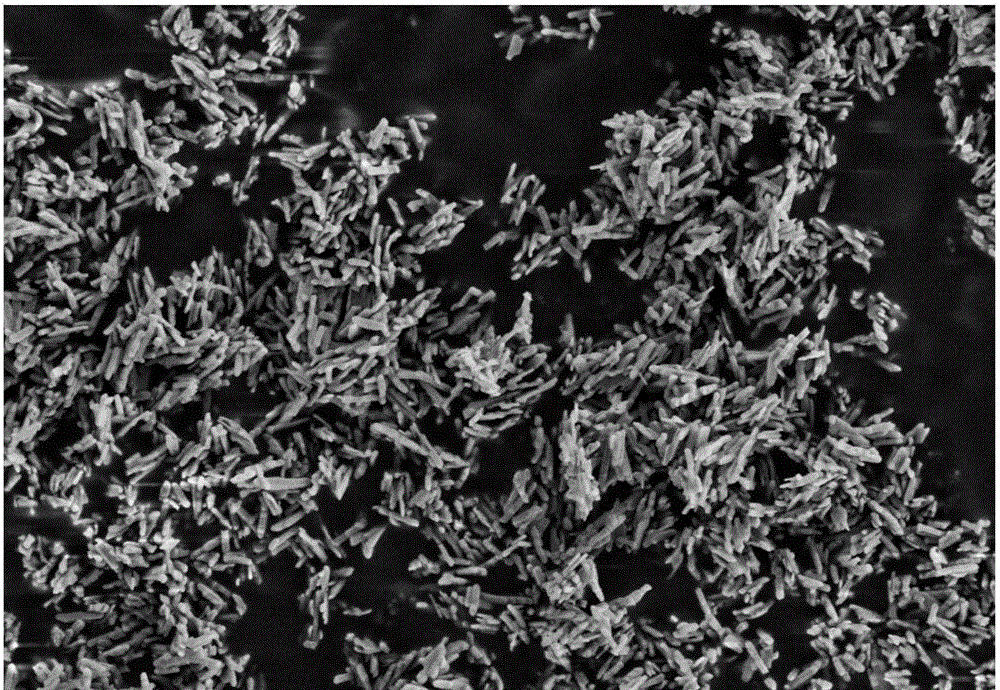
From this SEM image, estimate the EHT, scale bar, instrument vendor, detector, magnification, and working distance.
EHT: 3 kV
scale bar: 200 nm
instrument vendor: Zeiss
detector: SE2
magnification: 10 K X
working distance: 6.1 mm